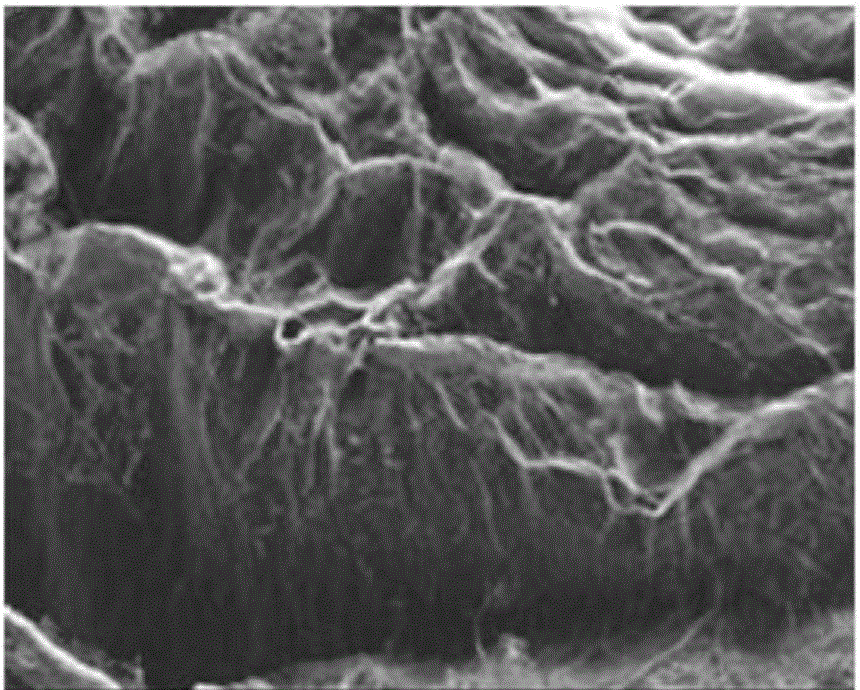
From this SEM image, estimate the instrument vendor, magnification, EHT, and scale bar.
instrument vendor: Zeiss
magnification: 0.1 K X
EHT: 15 kV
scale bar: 100000 nm